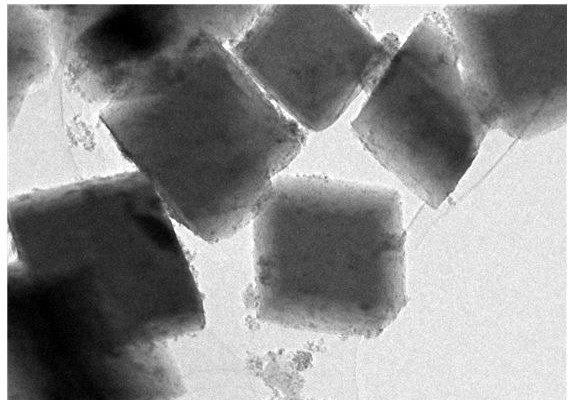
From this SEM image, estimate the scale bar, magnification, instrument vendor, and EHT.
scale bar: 500 nm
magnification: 10 K X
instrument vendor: JEOL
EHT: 200 kV